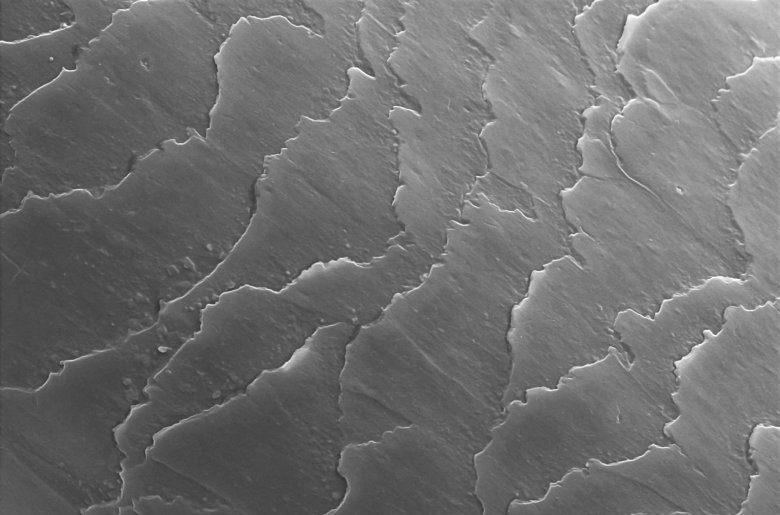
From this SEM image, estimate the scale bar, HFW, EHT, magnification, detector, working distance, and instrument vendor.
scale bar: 10000 nm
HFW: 63.5 µm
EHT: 15 kV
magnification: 2 K X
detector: ETD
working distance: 8 mm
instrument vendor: Thermo